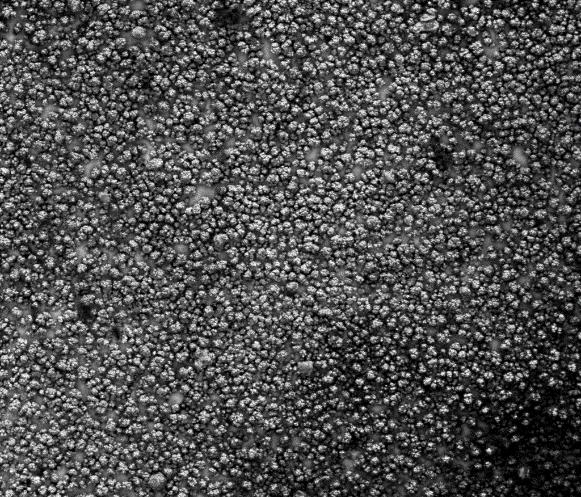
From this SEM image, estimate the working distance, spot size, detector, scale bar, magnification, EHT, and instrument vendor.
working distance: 10.9 mm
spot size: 5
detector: Mix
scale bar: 300000 nm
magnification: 0.2 K X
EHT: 20 kV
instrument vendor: FEI